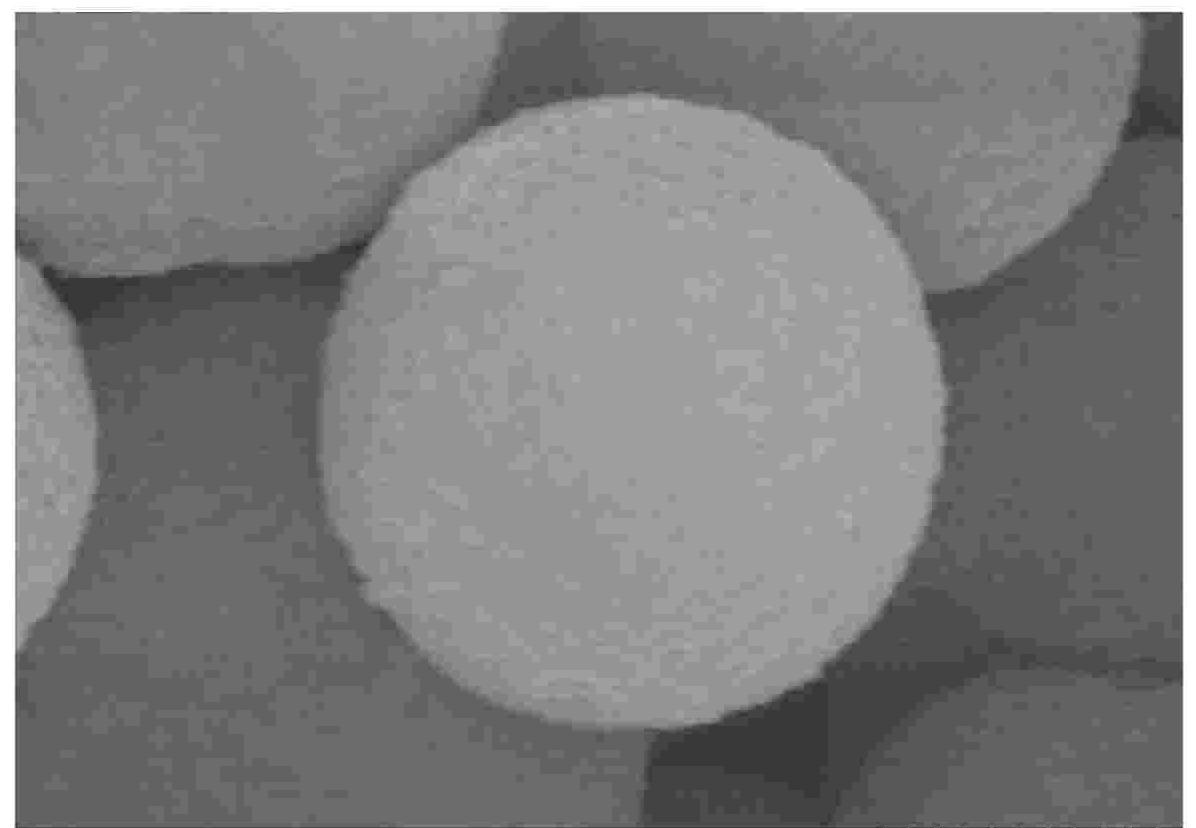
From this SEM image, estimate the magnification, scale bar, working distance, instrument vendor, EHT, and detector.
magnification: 60 K X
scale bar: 500 nm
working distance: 11.8 mm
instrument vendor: Hitachi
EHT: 15 kV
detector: SE(M)